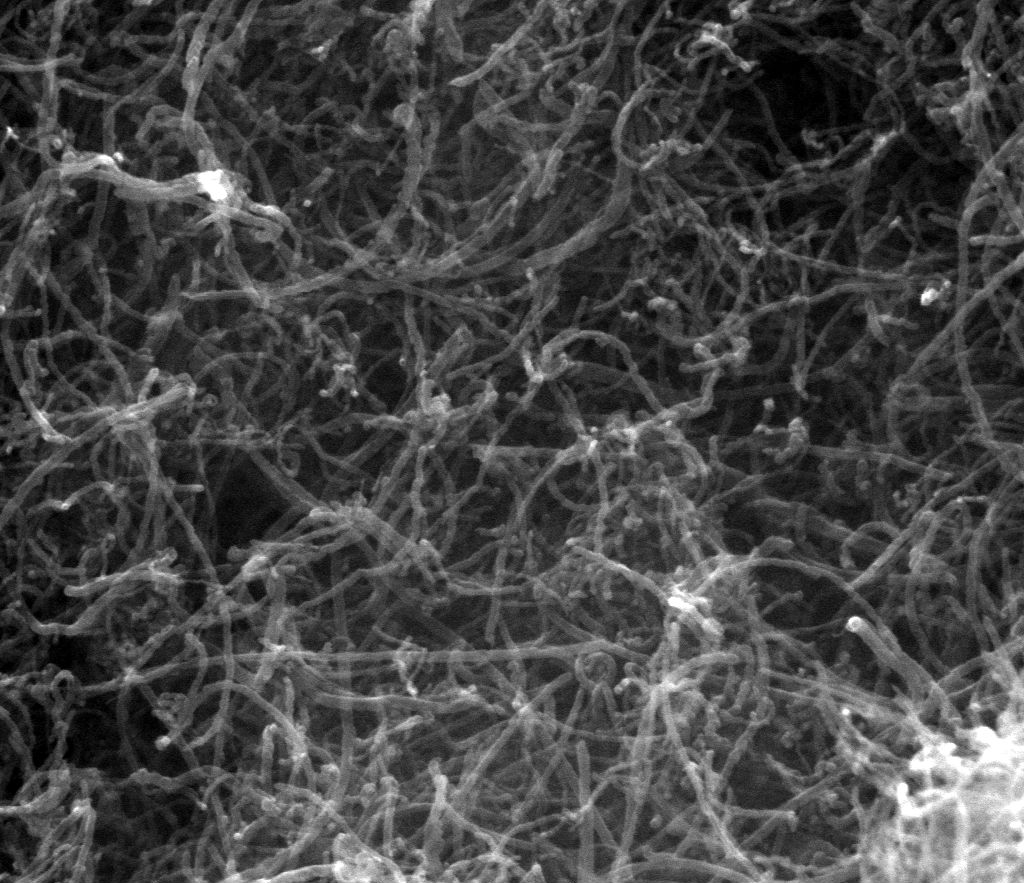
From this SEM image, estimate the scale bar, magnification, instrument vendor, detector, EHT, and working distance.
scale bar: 1000 nm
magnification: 80 K X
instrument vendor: FEI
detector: SE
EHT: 20 kV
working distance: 11.9 mm